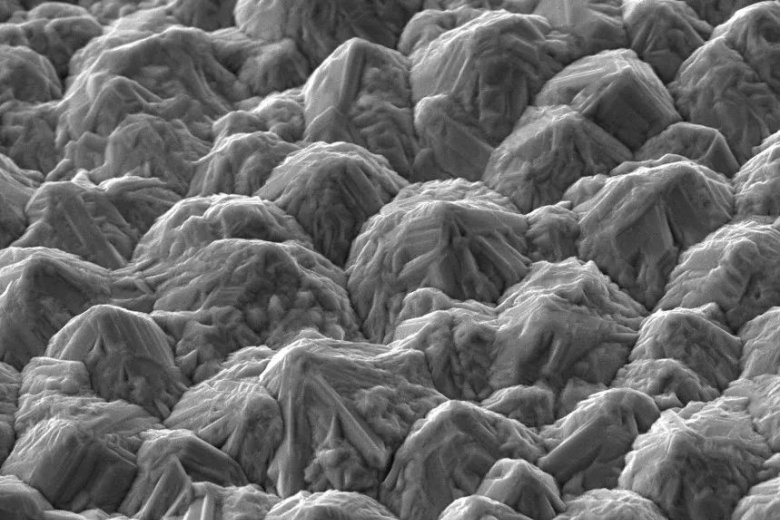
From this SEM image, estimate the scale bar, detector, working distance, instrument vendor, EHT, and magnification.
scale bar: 4000 nm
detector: SE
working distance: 15 mm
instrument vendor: other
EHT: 10 kV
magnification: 8 K X